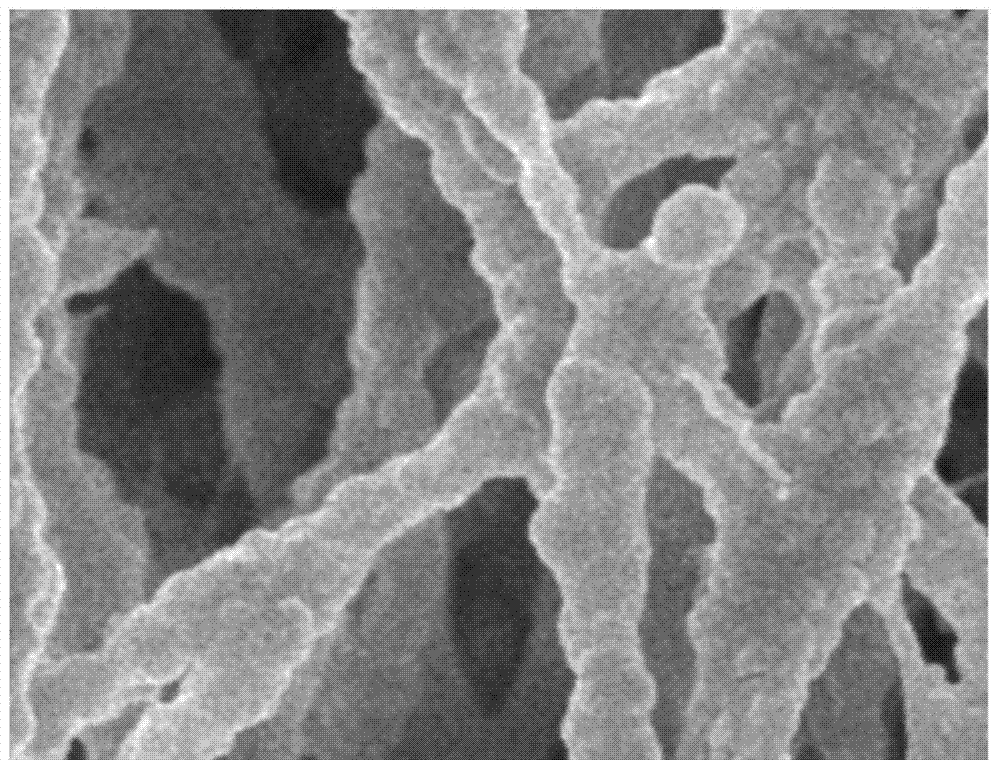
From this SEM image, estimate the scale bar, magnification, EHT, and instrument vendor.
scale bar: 1000 nm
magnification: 10 K X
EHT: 5 kV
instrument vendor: JEOL